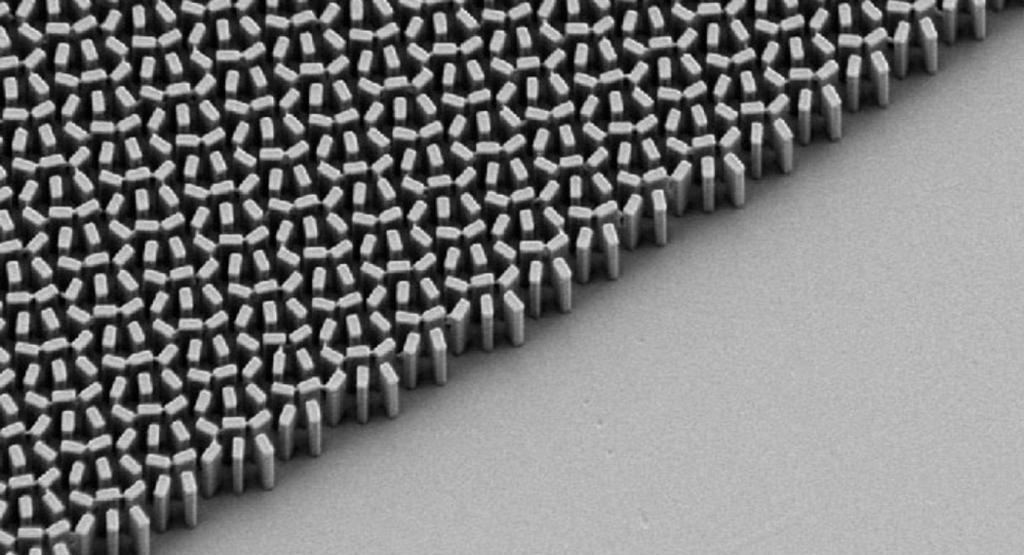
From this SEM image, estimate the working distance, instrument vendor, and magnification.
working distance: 6.7 mm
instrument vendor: Zeiss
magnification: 22.81 K X